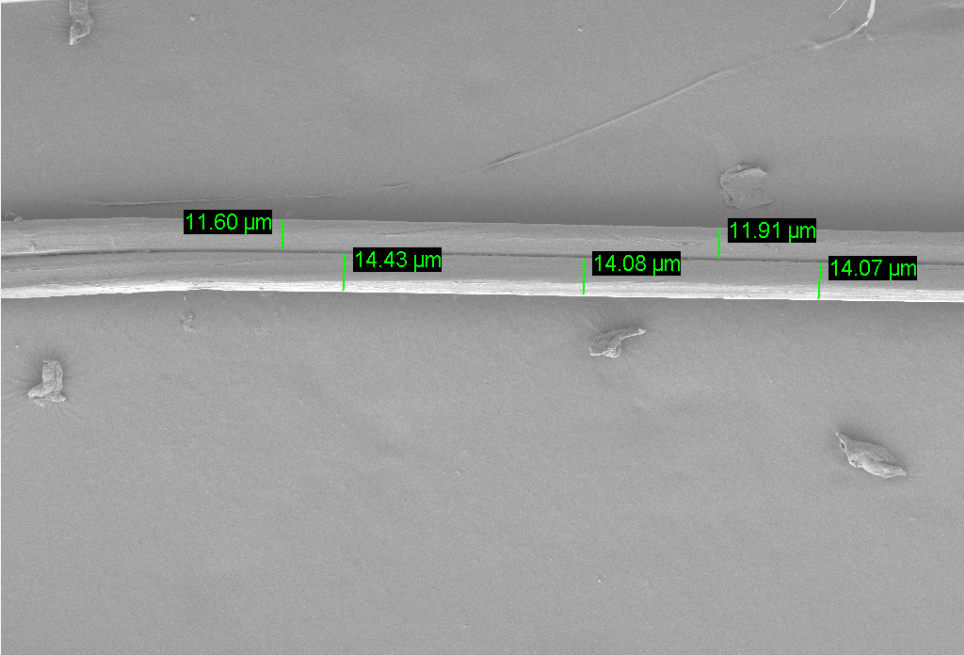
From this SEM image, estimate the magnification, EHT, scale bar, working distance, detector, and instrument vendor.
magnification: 0.309 K X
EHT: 2 kV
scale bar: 20000 nm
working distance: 8.4 mm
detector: SE2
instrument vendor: Zeiss